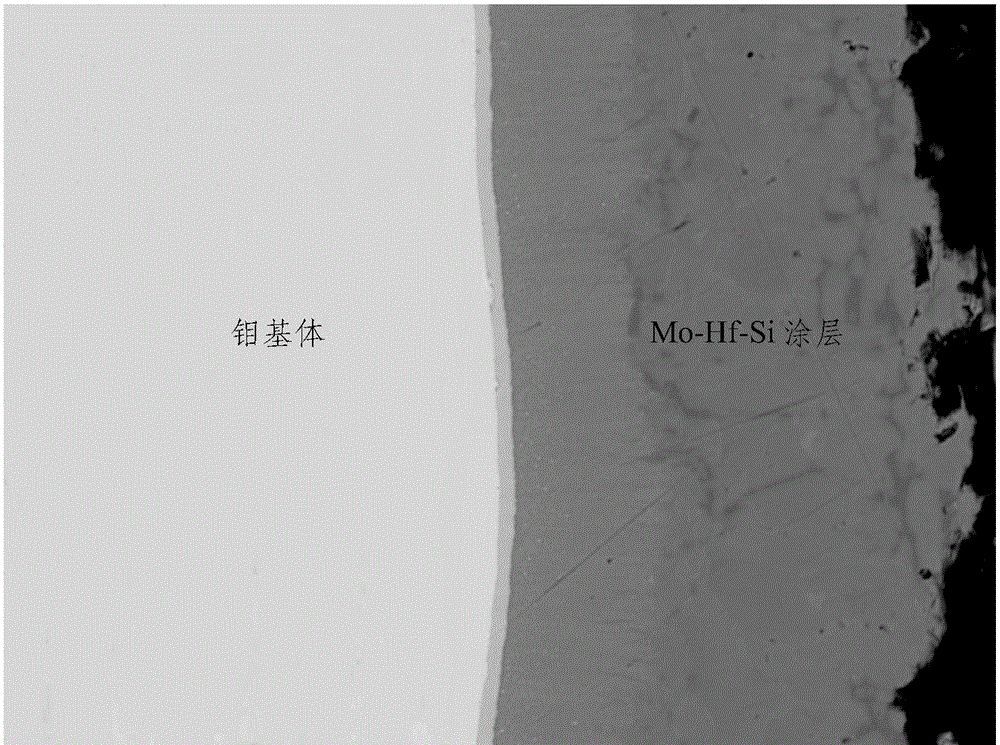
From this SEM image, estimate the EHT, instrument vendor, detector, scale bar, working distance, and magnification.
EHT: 20 kV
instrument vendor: JEOL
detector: COMPO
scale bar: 10000 nm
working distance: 10.9 mm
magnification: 0.5 K X